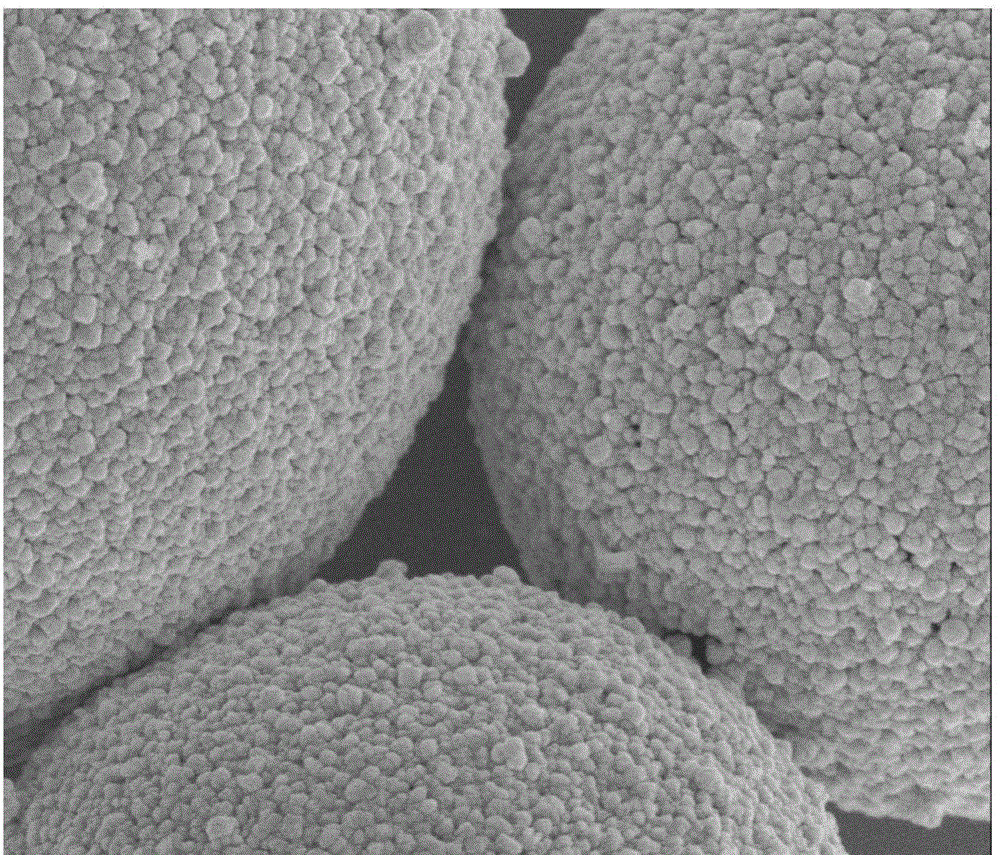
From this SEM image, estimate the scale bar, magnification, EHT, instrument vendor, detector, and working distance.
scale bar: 1000 nm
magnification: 30 K X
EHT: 5 kV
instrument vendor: FEI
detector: TLD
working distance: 4.8 mm